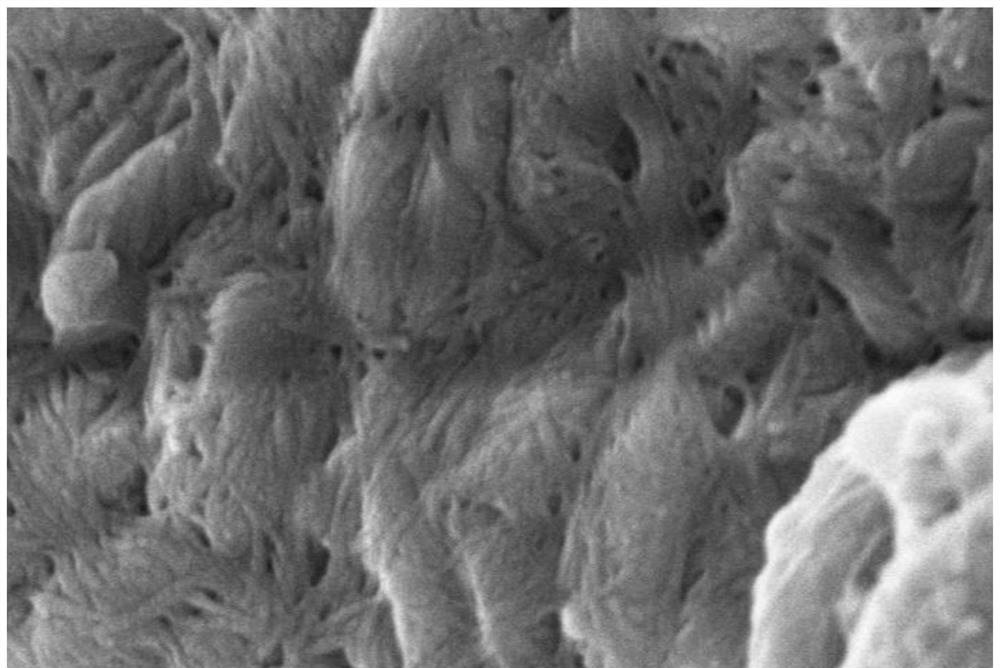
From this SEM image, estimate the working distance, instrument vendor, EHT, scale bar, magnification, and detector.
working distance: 5.9 mm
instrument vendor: Hitachi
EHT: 3 kV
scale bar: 500 nm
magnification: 100 K X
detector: SE(UL)